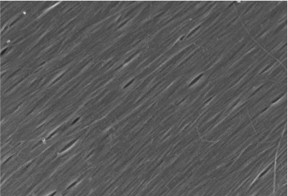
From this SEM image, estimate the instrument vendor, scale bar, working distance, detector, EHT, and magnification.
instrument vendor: Hitachi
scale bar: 500 nm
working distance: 1.5 mm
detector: SE(T)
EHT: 1 kV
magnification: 100 K X